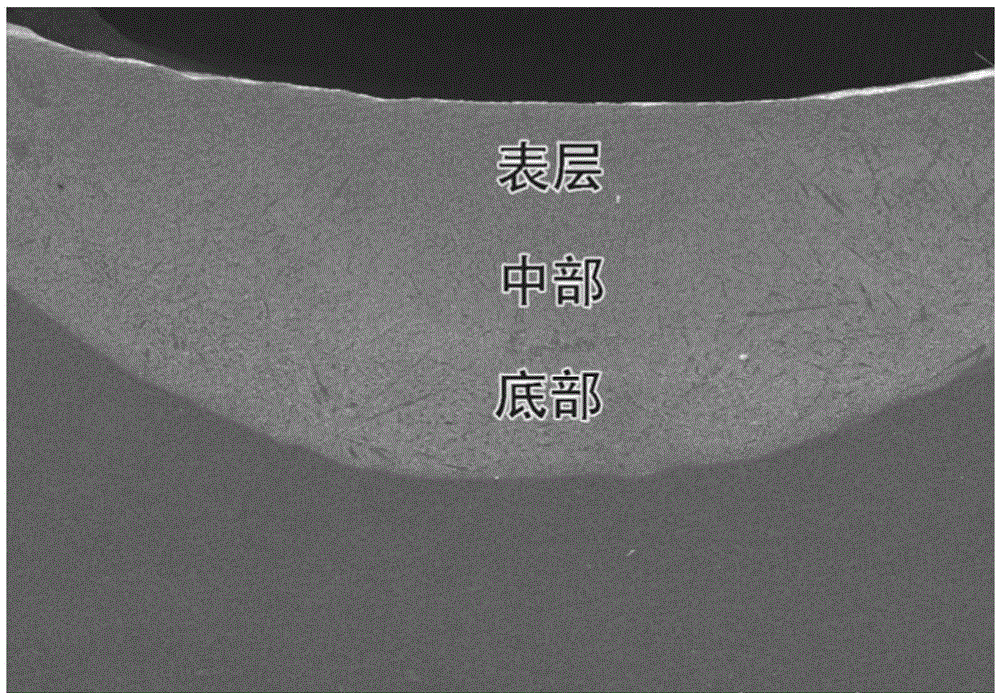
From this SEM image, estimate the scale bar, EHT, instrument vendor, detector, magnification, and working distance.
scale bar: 1000000 nm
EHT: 20 kV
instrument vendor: Hitachi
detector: LM(UL)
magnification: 0.03 K X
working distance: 10.9 mm